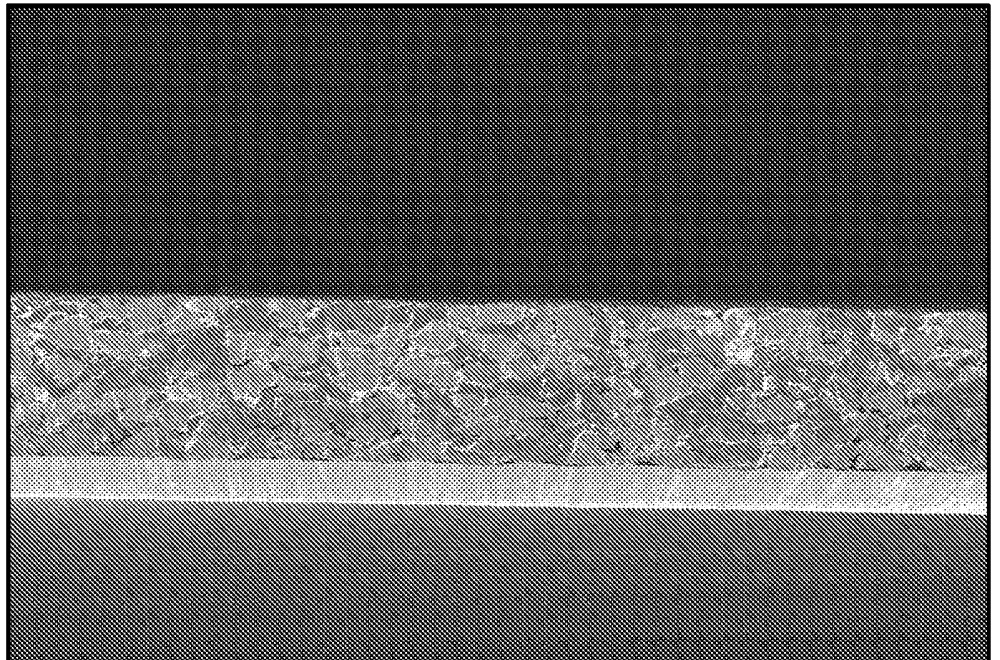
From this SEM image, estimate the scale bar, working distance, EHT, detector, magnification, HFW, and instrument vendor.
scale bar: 20000 nm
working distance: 4 mm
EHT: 1 kV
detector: InLens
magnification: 0.5 K X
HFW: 228.7 µm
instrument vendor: Zeiss